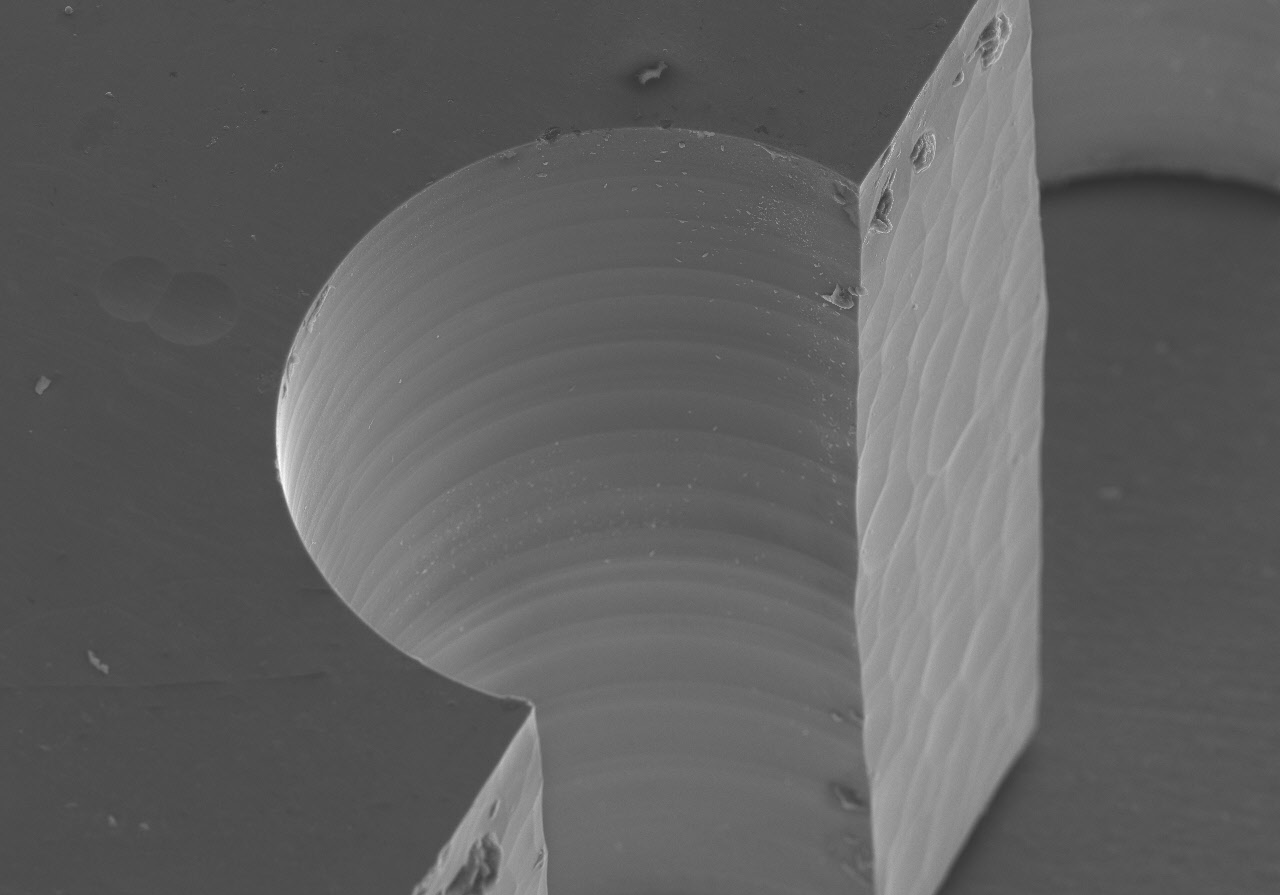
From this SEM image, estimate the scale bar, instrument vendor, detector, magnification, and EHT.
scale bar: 200000 nm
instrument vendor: Hitachi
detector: SE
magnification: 0.21 K X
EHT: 15 kV